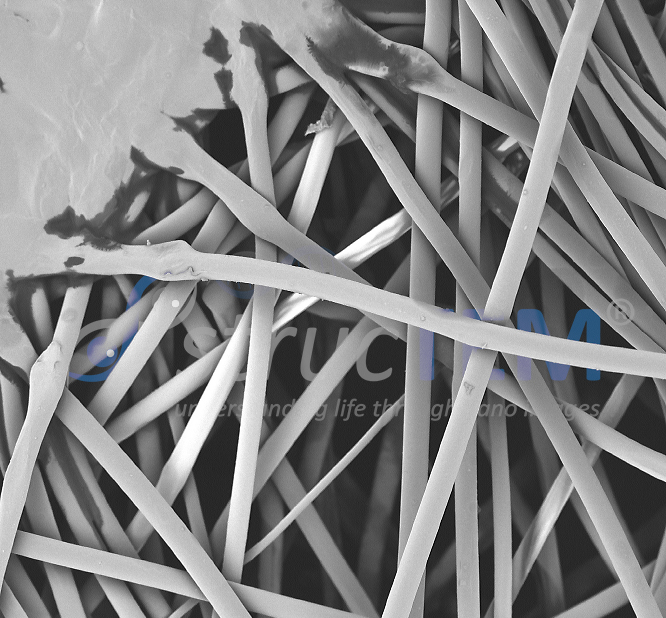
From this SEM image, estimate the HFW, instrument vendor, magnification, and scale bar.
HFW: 537 µm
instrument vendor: Phenom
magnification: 0.5 K X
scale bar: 100000 nm